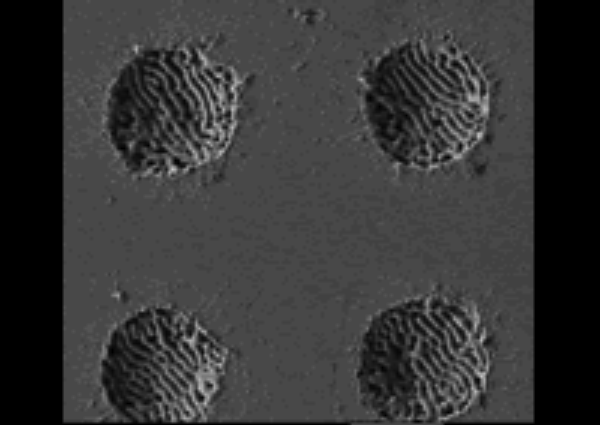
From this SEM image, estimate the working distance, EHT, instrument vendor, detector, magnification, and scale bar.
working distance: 5.7 mm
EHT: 2 kV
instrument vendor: JEOL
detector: LEI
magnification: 2 K X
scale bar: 10000 nm